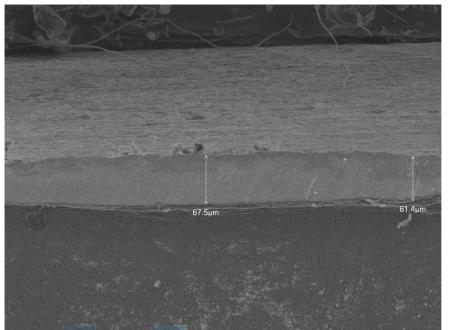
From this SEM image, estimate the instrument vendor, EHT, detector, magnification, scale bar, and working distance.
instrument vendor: JEOL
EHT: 15 kV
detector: LEI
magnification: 0.2 K X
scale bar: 100000 nm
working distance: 8 mm